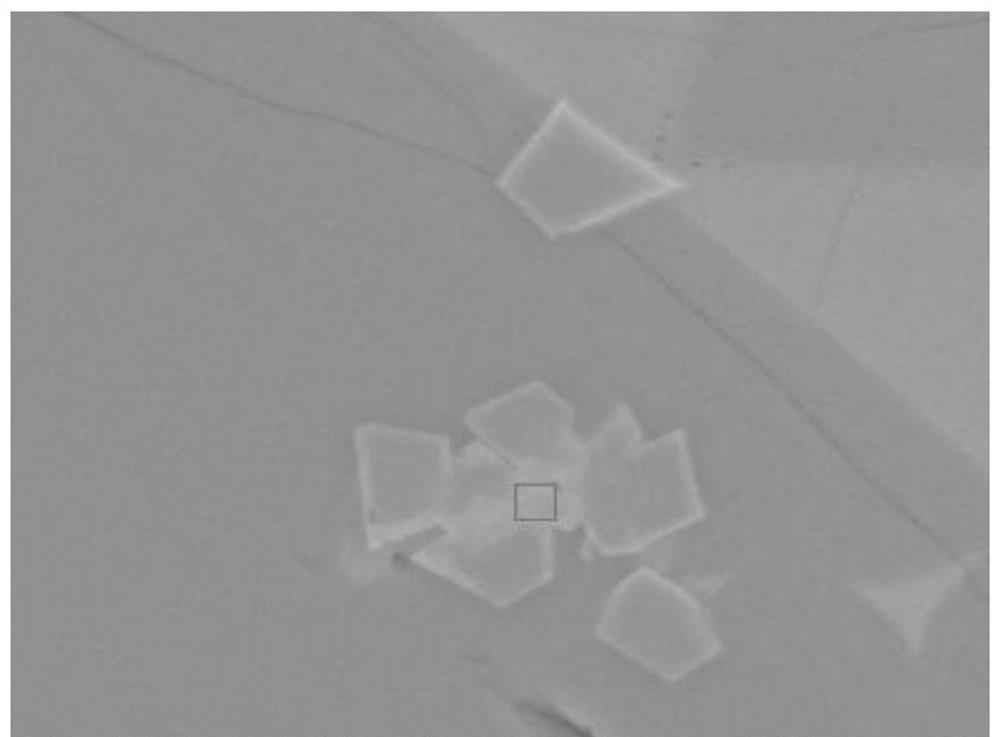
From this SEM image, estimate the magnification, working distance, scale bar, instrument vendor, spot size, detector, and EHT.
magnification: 5 K X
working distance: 13.2 mm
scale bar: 20000 nm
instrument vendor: FEI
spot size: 4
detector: BSED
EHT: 20 kV